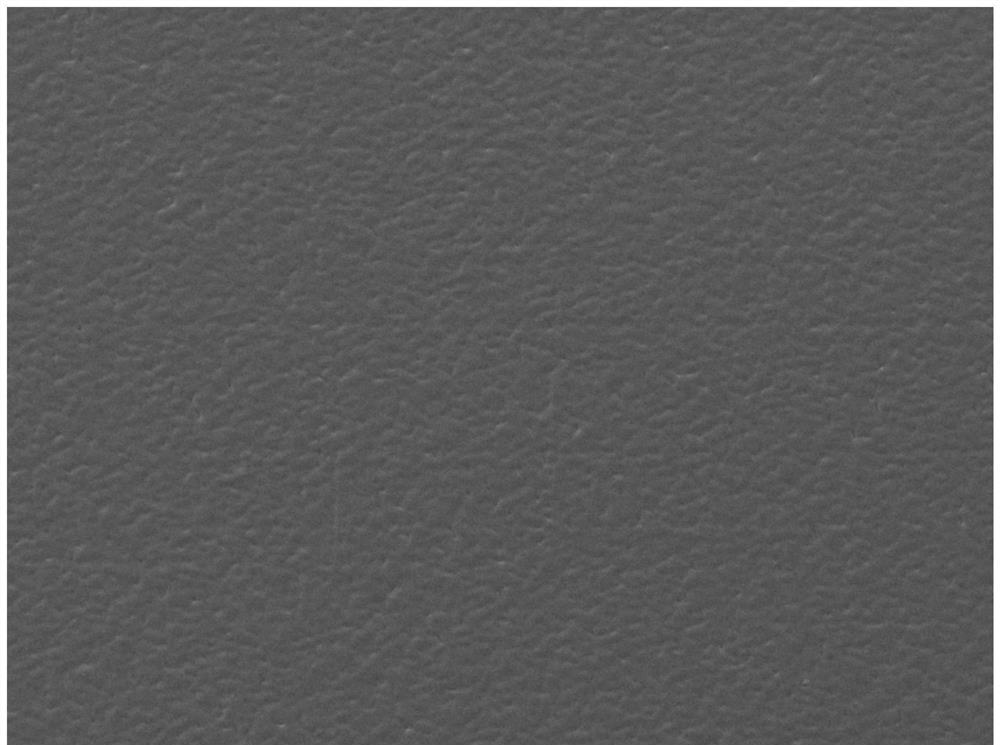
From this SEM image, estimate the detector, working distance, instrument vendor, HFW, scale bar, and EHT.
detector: SE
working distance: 8.52 mm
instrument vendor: Tescan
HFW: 1040 µm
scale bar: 200000 nm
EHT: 30 kV